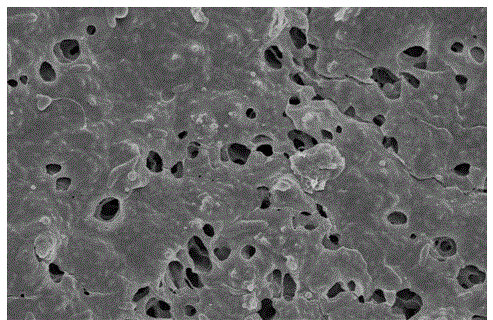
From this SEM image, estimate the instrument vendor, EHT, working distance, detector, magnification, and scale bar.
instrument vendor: Hitachi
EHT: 5 kV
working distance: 6.3 mm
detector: SE(M)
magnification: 5 K X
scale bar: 10000 nm